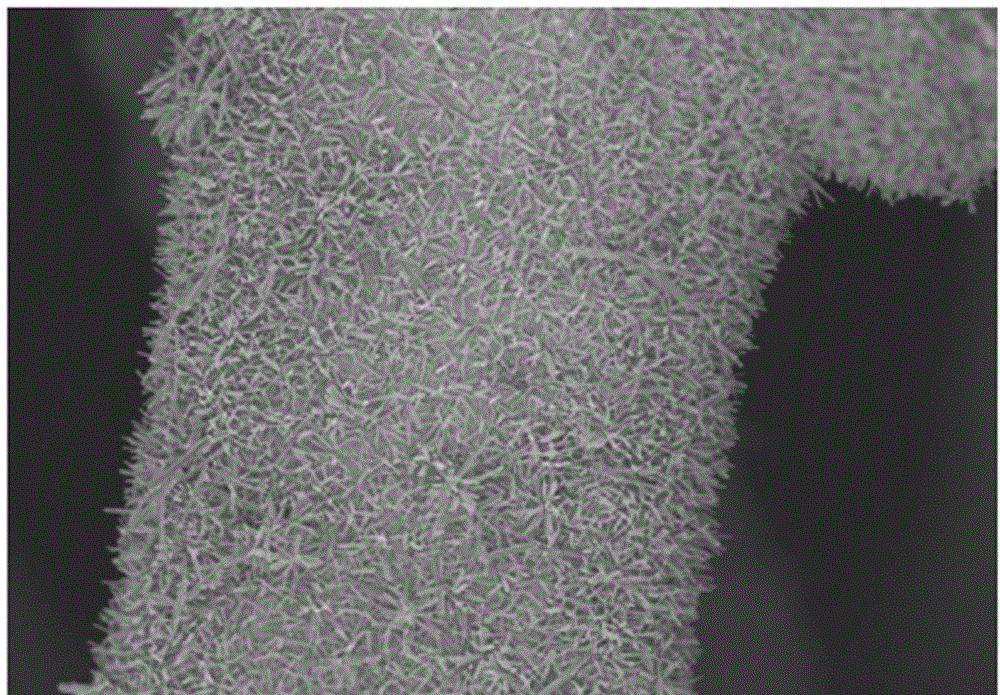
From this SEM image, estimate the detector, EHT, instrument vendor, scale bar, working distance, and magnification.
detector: SE(M)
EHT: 3 kV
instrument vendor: Hitachi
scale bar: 50000 nm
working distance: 4.7 mm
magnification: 1 K X